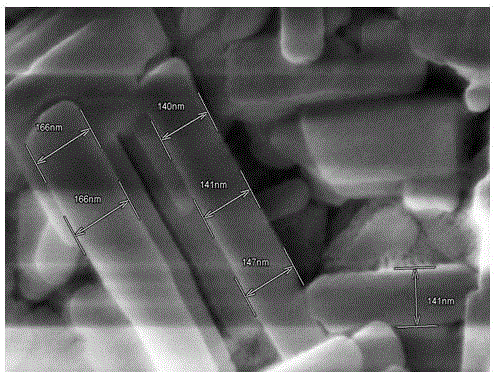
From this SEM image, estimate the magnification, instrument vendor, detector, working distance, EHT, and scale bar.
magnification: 100 K X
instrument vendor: Hitachi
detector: SE(U)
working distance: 9 mm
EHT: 15 kV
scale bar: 500 nm